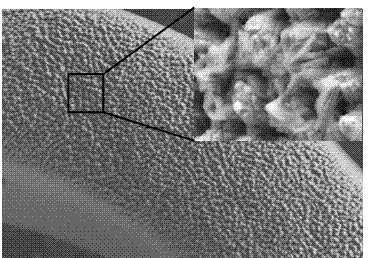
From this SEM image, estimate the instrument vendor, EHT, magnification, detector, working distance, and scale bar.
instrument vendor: Hitachi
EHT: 15 kV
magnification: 1 K X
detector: SE(L)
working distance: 7.8 mm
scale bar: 50000 nm